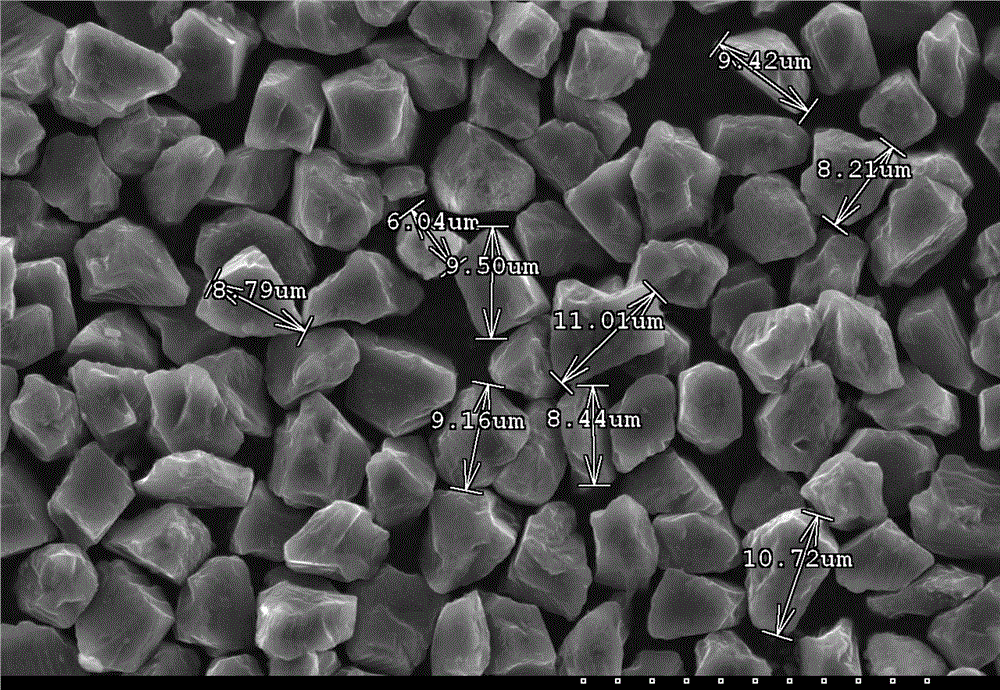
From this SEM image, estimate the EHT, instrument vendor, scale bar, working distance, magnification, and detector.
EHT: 20 kV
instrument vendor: Hitachi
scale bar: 30000 nm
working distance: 9.7 mm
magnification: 1.5 K X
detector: SE